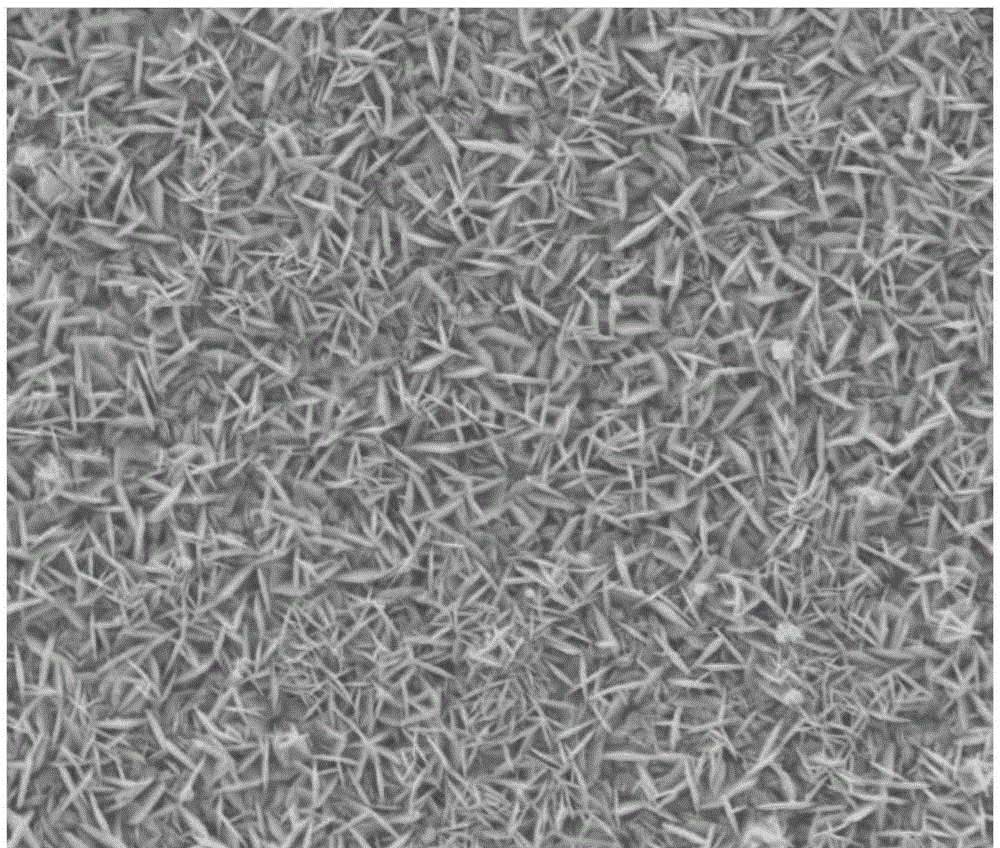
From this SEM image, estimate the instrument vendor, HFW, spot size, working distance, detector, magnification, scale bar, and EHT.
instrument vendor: FEI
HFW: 59.7 µm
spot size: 3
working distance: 9.6 mm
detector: ETD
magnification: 5 K X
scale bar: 20000 nm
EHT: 20 kV